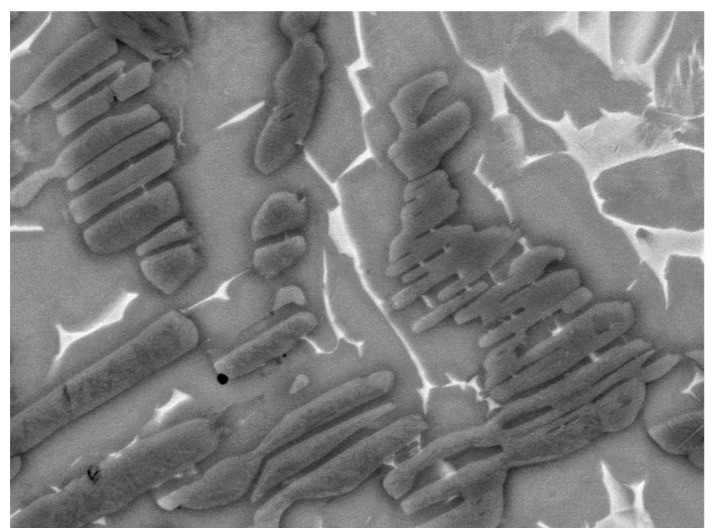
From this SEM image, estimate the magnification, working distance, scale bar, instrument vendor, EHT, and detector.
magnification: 4.5 K X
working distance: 10.7 mm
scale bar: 5000 nm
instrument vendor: JEOL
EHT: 15 kV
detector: BED-C